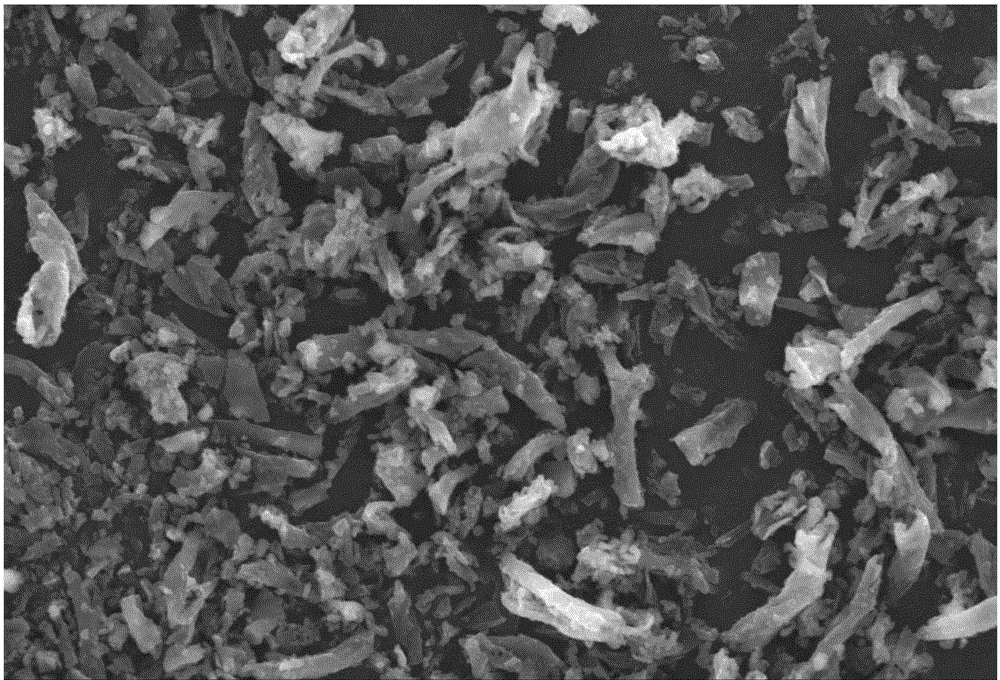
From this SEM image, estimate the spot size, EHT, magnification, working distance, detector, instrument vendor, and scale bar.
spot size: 30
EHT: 20 kV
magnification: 1 K X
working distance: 12 mm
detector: SEI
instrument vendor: JEOL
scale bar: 10000 nm